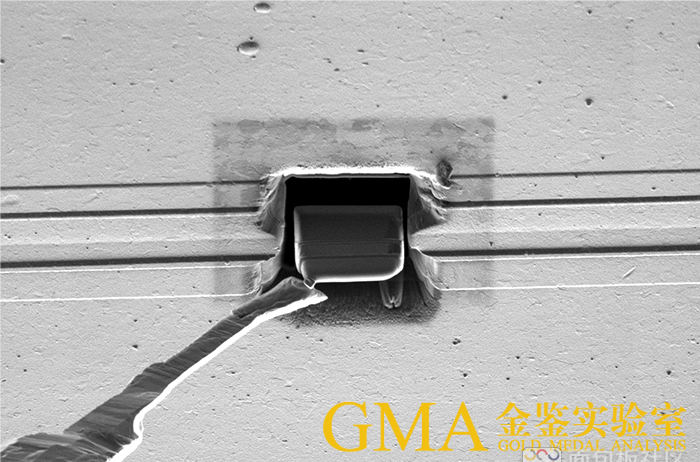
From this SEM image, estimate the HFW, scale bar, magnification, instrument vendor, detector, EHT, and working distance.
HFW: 63.5 µm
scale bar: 20000 nm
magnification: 2 K X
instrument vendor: FEI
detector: ETD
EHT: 30 kV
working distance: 19 mm